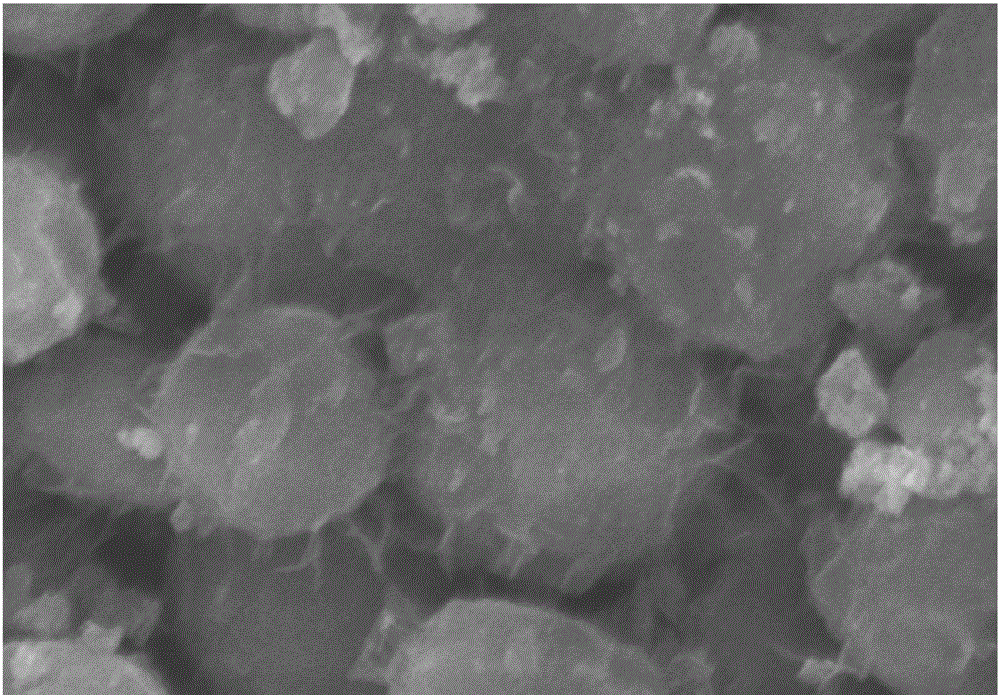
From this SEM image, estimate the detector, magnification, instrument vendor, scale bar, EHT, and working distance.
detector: SE(M)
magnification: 100 K X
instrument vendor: Hitachi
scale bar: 500 nm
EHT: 5 kV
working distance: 8.8 mm